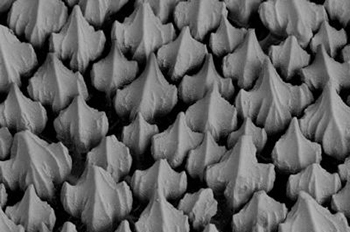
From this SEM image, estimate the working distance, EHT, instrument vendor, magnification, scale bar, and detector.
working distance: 43 mm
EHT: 20 kV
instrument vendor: Zeiss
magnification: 0.144 K X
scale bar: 100000 nm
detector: RBSD